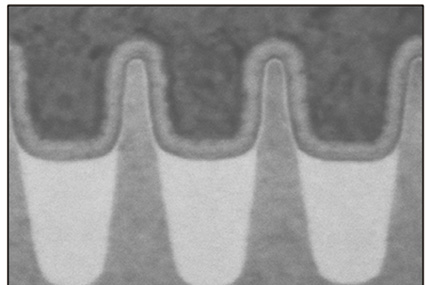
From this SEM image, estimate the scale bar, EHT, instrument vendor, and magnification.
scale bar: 50 nm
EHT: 30 kV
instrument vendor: JEOL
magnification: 700 K X